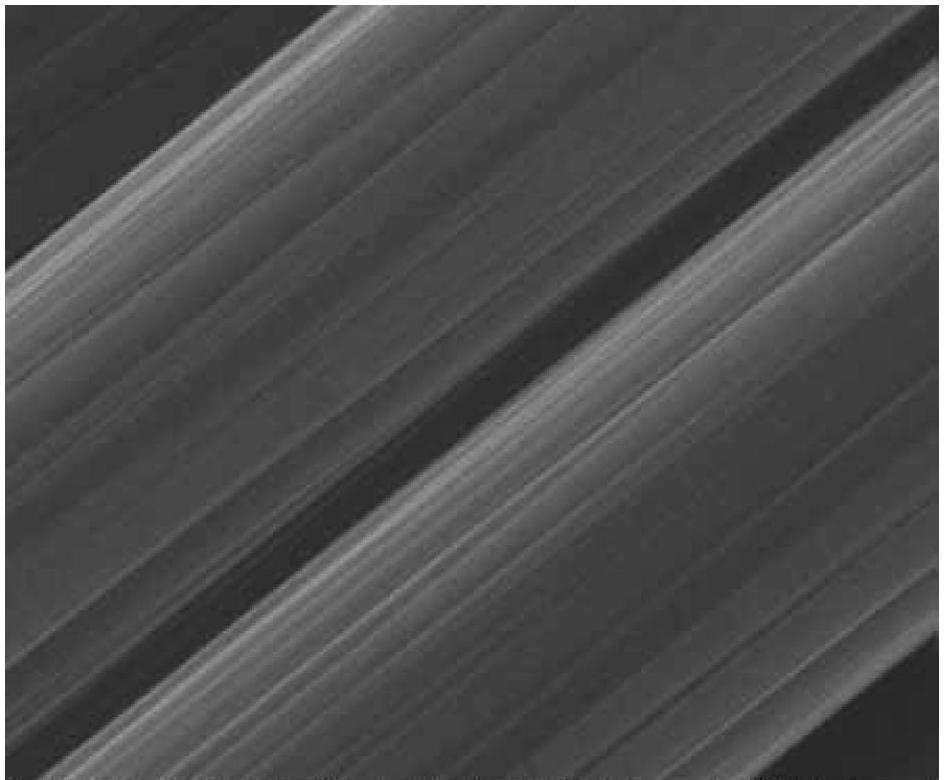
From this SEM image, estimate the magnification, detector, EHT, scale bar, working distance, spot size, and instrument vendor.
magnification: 10 K X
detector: ETD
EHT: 12.5 kV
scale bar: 2000 nm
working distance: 10.4 mm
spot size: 3.5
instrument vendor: FEI